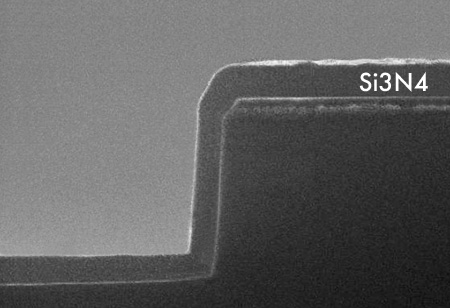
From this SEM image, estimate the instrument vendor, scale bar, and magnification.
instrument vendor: JEOL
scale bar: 1000 nm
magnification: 15 K X